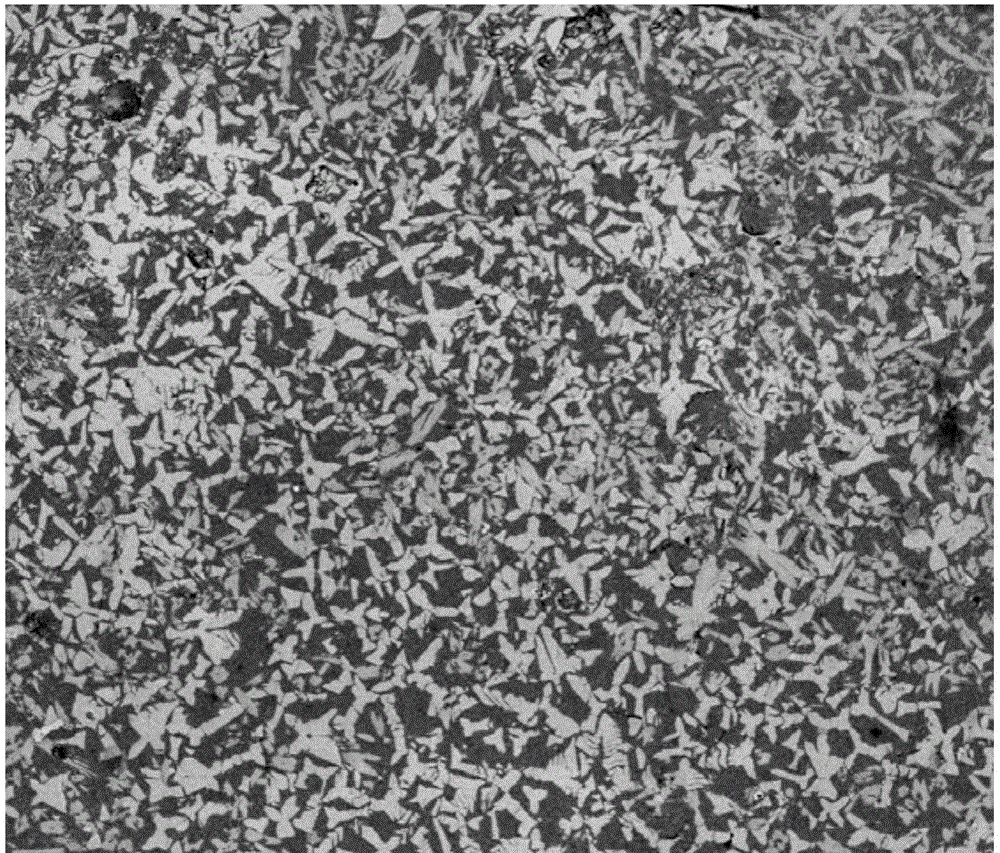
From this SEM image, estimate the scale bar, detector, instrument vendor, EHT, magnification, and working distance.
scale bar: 50000 nm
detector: CBS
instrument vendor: FEI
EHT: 5 kV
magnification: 1 K X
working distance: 5.3 mm